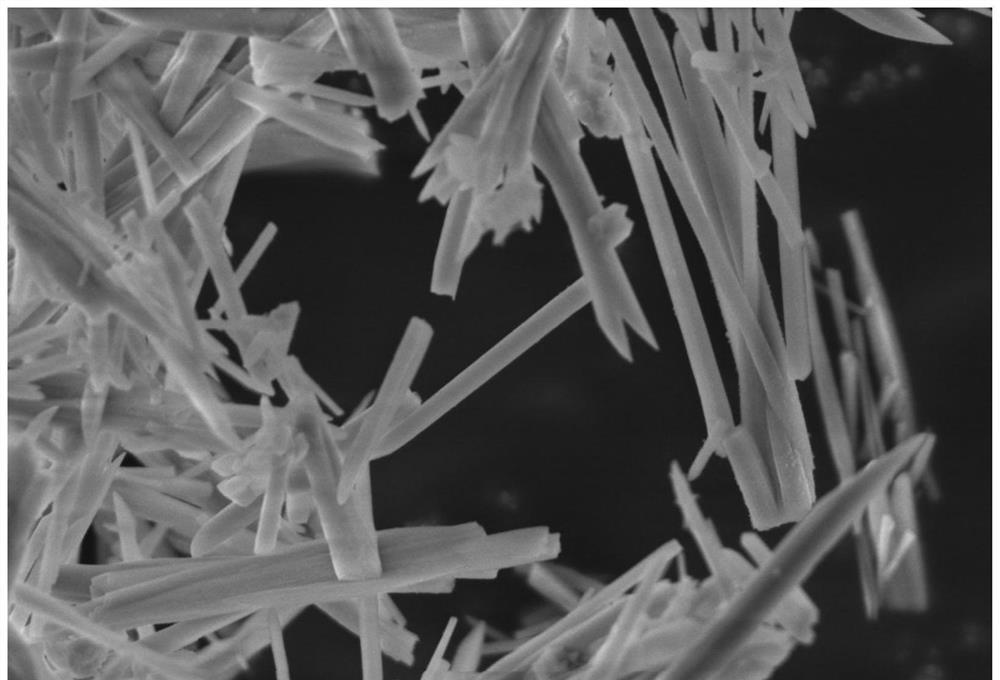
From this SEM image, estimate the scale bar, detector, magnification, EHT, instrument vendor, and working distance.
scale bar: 400 nm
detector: InLens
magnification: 20 K X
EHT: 5 kV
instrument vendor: Zeiss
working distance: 7.1 mm